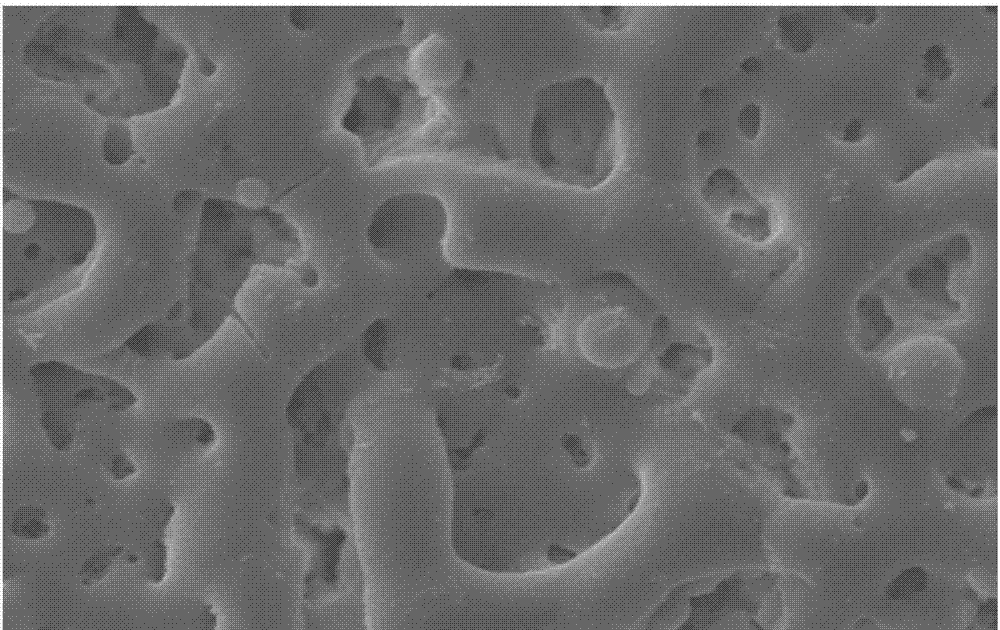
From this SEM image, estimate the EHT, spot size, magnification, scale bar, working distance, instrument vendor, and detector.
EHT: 15 kV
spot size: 4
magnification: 3 K X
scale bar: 5000 nm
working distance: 15 mm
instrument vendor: FEI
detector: SE